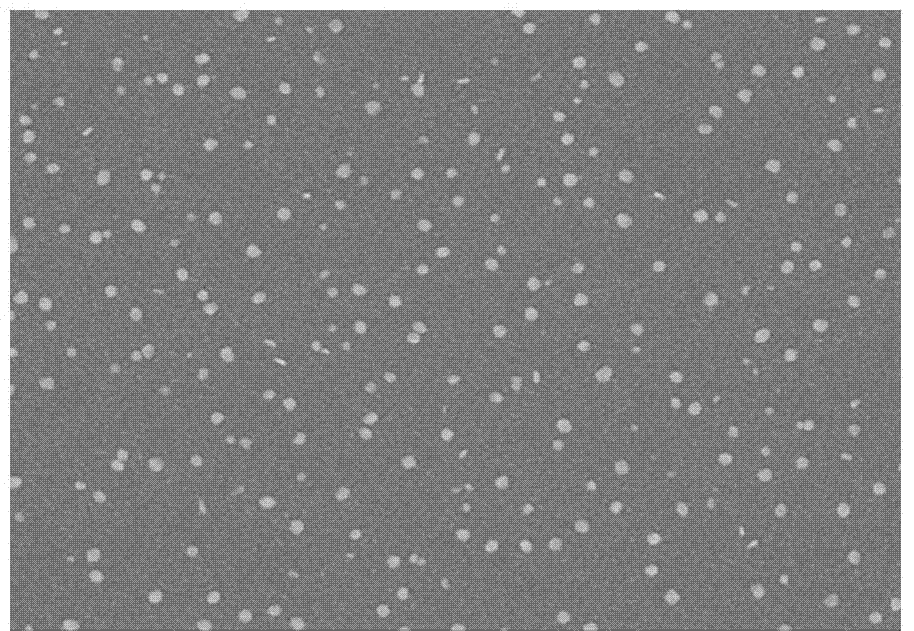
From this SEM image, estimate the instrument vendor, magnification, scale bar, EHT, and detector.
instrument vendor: Hitachi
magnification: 30 K X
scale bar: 1000 nm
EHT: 8 kV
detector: SE(M)